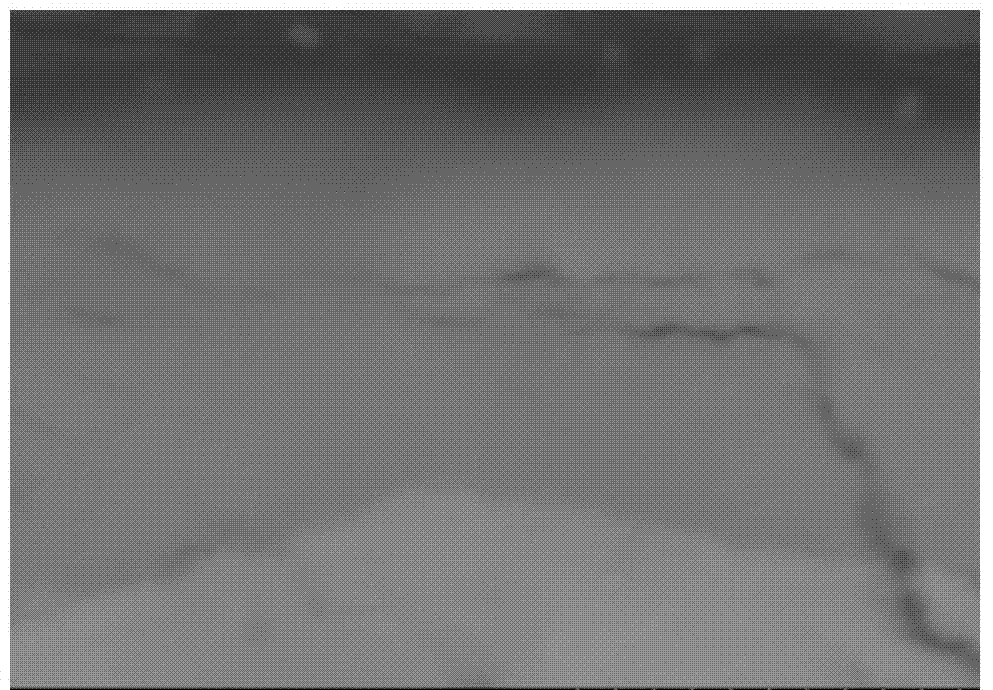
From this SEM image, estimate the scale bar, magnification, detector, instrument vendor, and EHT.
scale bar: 5000 nm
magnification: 10 K X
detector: BSECOMP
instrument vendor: Hitachi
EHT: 20 kV